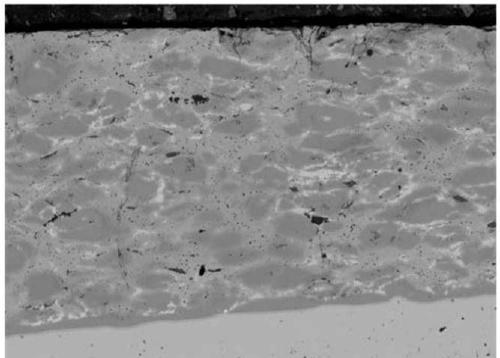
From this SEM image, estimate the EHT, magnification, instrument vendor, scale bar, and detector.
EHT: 15 kV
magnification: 0.15 K X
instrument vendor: JEOL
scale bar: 200000 nm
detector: COMPO